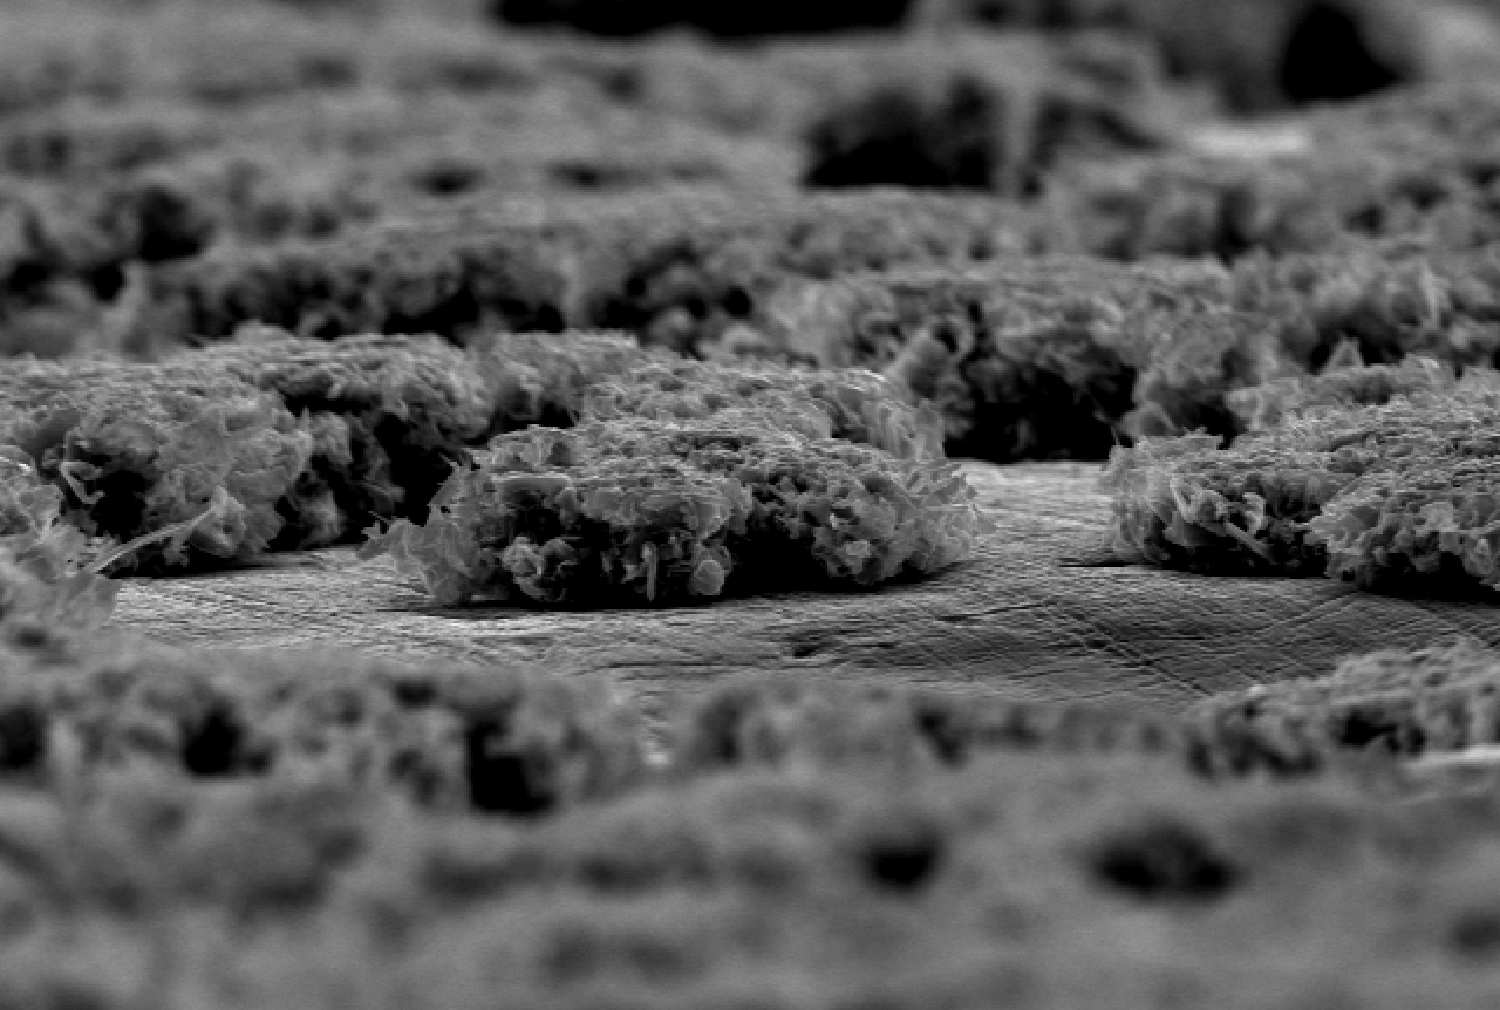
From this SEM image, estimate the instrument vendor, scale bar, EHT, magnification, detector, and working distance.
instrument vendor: Hitachi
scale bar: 100000 nm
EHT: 20 kV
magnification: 0.27 K X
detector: SE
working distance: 25.2 mm